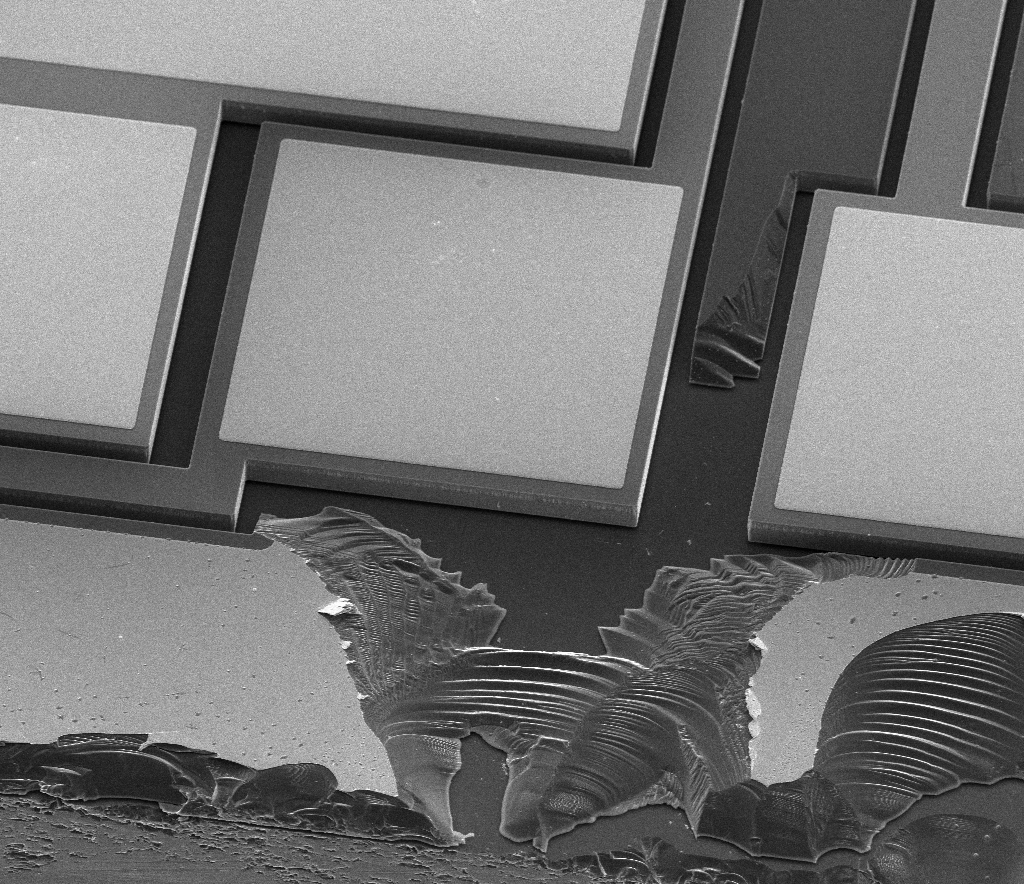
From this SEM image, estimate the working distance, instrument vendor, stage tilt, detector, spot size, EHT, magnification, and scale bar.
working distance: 20.4 mm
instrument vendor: FEI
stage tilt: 41°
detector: ETD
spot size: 2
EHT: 10 kV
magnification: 0.554 K X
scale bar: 100000 nm